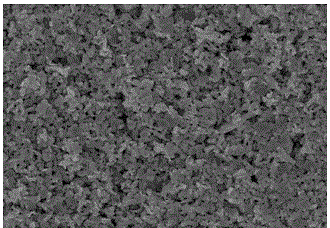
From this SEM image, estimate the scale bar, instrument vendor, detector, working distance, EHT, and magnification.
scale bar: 20000 nm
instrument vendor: Hitachi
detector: SE(U)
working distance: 7.7 mm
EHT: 15 kV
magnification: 2 K X